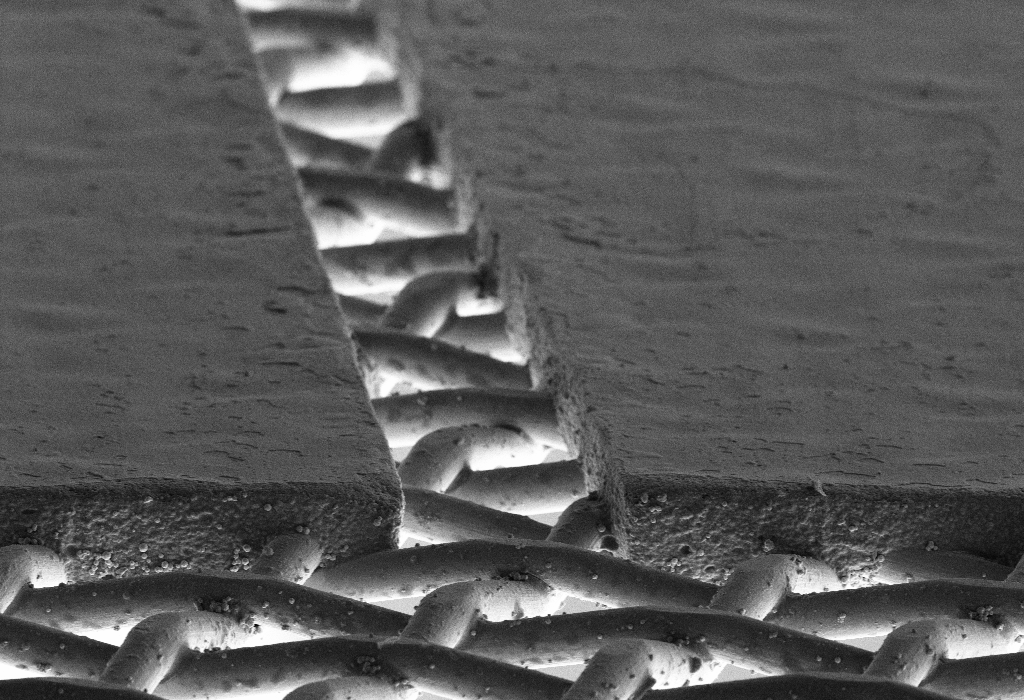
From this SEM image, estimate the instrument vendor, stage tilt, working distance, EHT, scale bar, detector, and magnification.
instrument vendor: Zeiss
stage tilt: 12.6°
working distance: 3.7 mm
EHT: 5 kV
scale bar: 20000 nm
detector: SE2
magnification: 0.5 K X